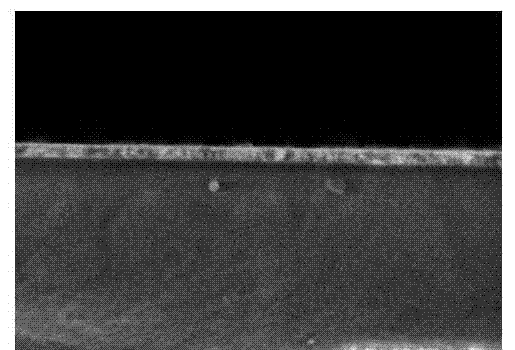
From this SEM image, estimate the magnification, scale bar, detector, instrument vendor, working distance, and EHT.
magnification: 70 K X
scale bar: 500 nm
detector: SE(U)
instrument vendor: Hitachi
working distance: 4.4 mm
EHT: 1 kV